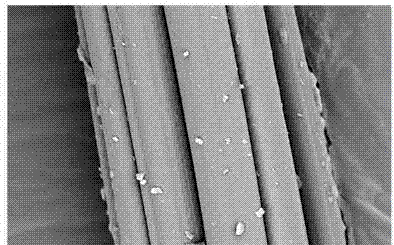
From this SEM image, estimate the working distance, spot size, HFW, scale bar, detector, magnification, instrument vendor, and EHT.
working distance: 6.3 mm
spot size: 2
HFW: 16.6 µm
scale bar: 5000 nm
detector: CBS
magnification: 25 K X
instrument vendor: FEI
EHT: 5 kV